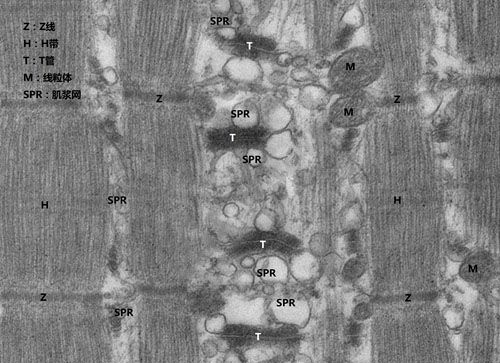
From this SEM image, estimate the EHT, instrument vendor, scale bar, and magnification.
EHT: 80 kV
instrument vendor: Hitachi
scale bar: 1000 nm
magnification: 8 K X